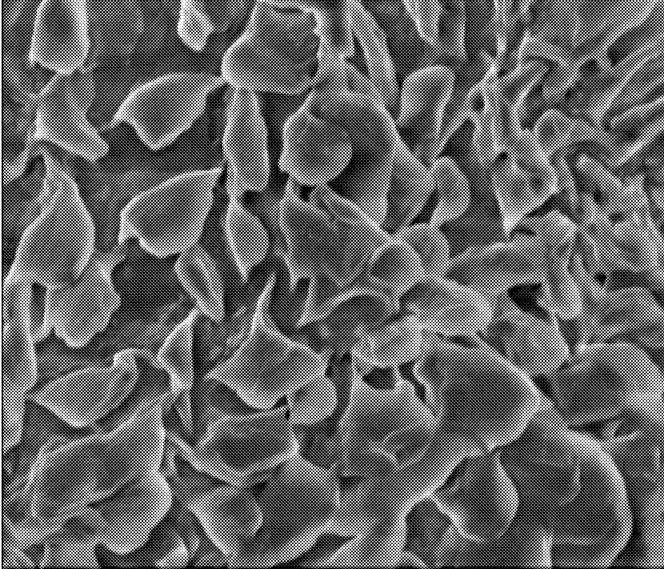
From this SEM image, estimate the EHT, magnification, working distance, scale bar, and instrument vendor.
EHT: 2 kV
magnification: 60 K X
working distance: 4.9 mm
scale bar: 3000 nm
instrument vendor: FEI